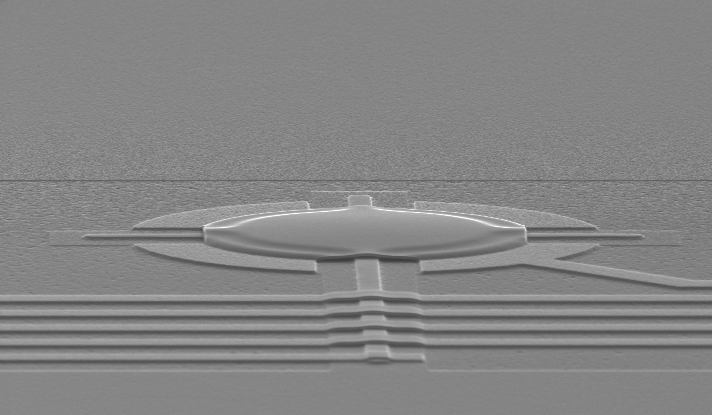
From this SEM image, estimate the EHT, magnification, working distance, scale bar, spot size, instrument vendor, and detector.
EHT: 30 kV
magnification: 4.259 K X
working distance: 7 mm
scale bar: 5000 nm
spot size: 4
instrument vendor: FEI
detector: SE